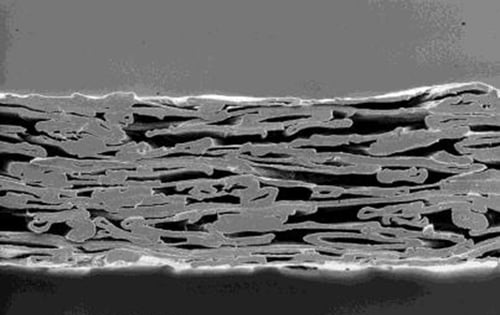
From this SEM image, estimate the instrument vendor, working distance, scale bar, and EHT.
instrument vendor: JEOL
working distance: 12 mm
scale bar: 10000 nm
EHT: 6 kV